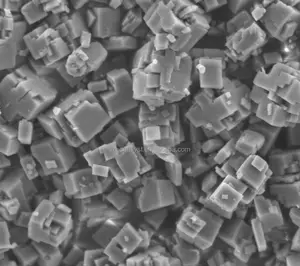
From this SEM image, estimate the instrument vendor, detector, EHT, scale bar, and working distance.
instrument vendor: JEOL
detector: LED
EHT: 3 kV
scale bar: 1000 nm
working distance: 8 mm